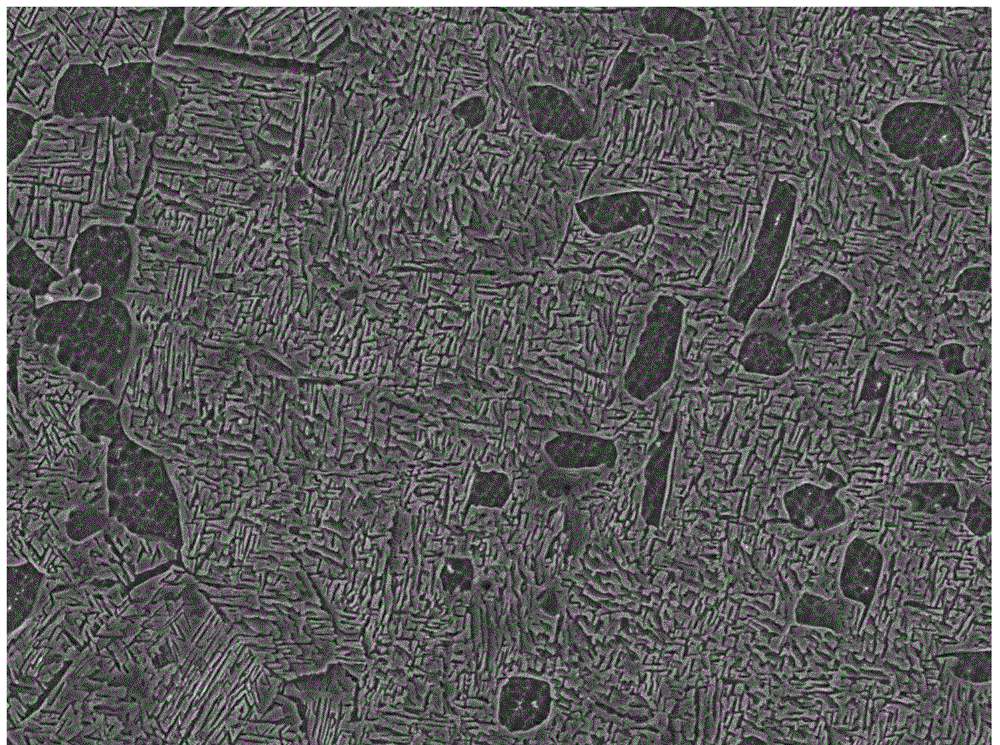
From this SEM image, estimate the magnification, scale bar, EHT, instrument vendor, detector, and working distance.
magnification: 5 K X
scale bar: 1000 nm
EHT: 5 kV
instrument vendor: JEOL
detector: SEI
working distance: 8.9 mm